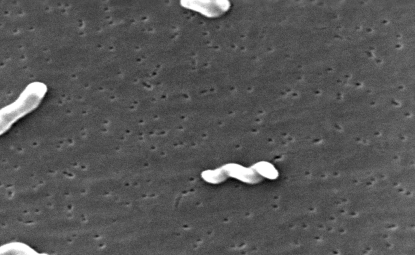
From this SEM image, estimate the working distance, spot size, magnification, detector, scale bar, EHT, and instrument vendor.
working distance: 10.5 mm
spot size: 4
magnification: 11.734 K X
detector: SE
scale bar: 2000 nm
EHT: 30 kV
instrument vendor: FEI